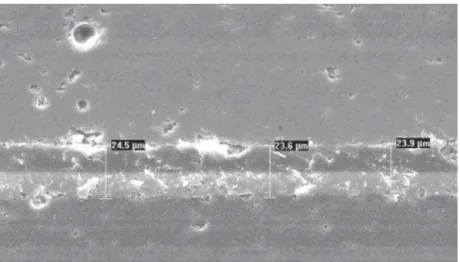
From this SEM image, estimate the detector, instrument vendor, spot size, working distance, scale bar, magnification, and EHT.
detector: SE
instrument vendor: FEI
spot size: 5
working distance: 10.6 mm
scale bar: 50000 nm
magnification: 0.5 K X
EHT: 20 kV